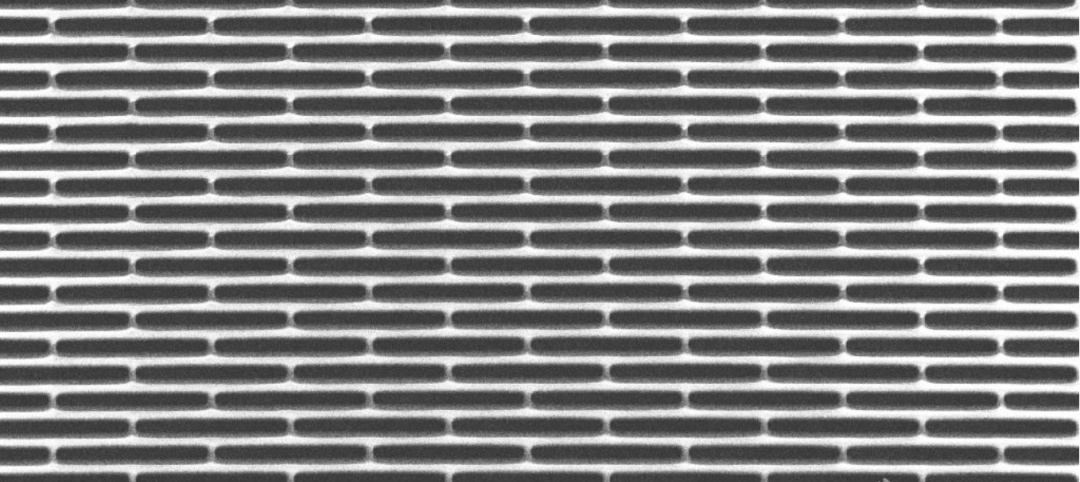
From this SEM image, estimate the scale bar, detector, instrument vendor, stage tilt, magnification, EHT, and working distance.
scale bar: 2000 nm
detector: SE2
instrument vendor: Zeiss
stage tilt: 0°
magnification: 1 K X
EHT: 3 kV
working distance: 15.2 mm